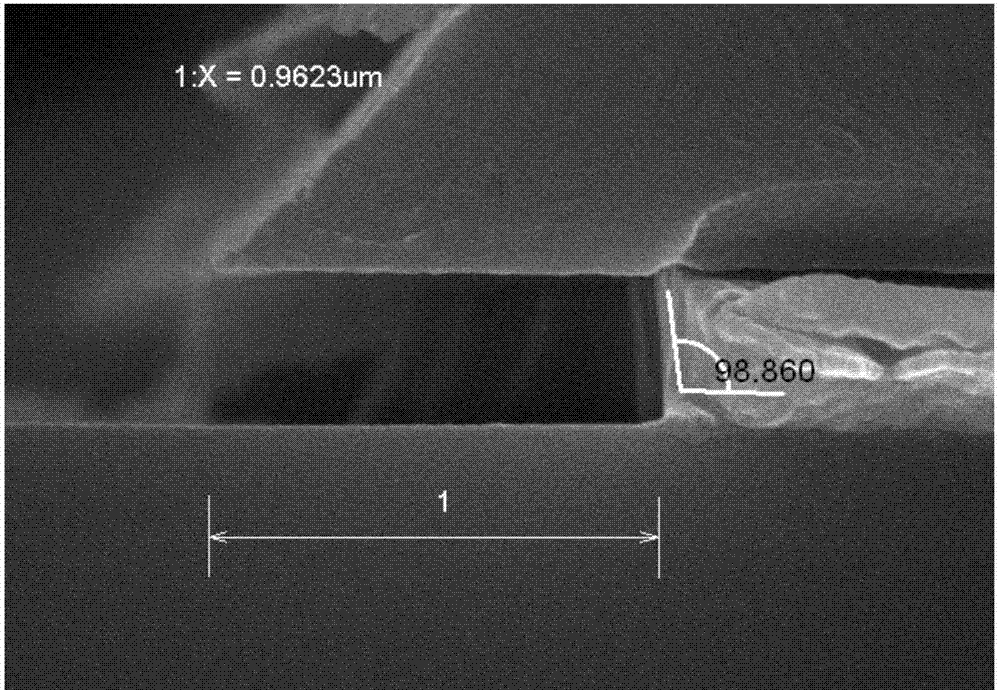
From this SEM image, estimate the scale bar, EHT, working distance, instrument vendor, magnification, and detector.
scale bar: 500 nm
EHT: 15 kV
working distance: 10.4 mm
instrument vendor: Hitachi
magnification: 60 K X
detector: SE(M)